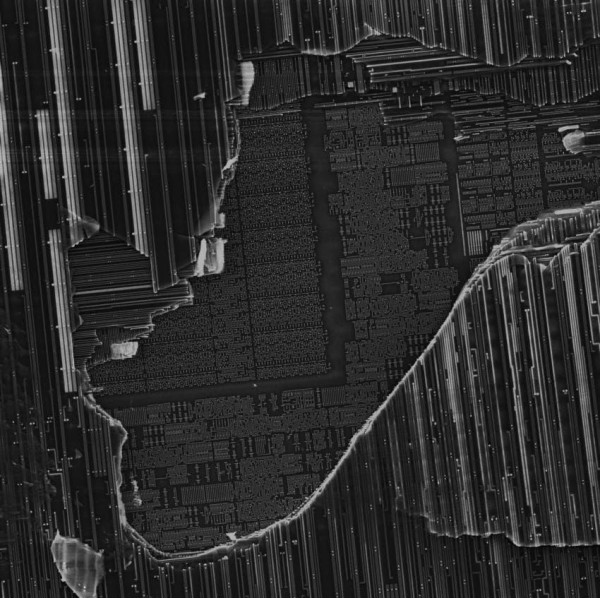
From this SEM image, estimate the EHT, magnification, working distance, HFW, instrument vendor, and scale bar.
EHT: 10 kV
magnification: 3.97 K X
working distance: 25.36 mm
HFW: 127 µm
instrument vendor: Tescan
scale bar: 20000 nm